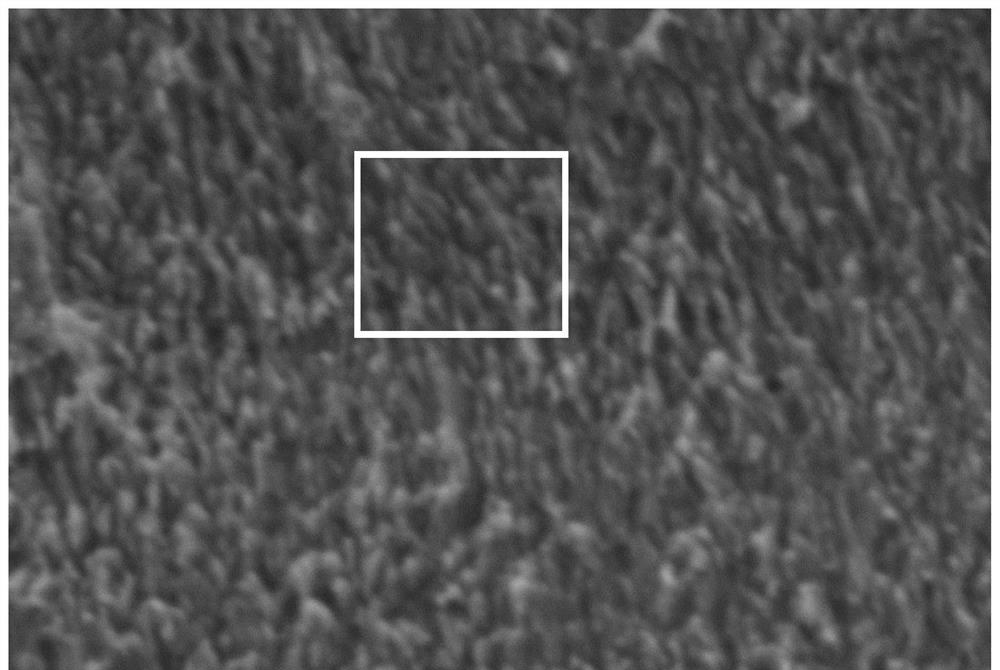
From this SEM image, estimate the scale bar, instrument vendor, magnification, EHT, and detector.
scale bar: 5000 nm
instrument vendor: JEOL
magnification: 5 K X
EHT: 15 kV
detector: SEI